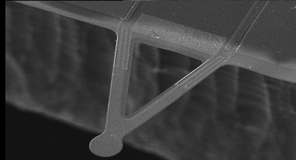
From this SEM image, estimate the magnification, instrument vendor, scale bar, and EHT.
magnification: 0.332 K X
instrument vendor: other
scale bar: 100000 nm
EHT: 25 kV